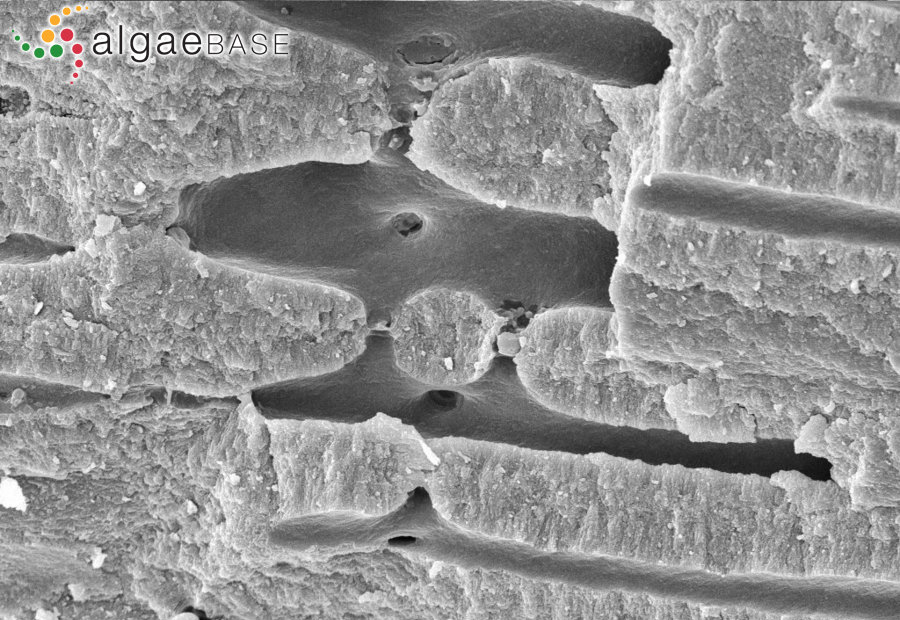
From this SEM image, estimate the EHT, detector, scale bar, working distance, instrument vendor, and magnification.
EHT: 11.73 kV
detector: SE1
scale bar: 2000 nm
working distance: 12 mm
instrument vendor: Zeiss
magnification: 3.46 K X